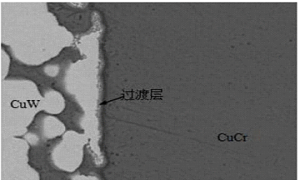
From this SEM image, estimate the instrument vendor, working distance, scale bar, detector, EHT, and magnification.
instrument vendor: JEOL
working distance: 15.1 mm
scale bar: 1000 nm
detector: COMPO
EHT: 20 kV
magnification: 3 K X